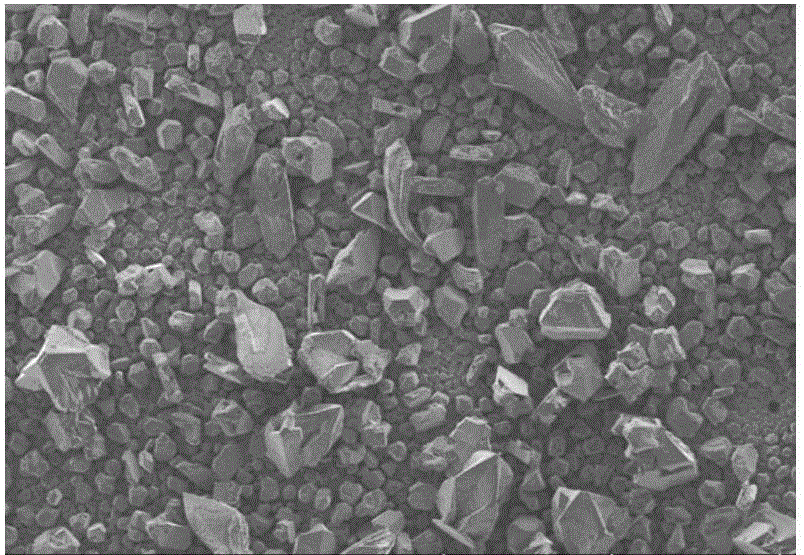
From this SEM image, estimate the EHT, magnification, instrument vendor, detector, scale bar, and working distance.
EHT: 5 kV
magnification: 0.18 K X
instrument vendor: Hitachi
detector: LM(UL)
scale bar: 300000 nm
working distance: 3.3 mm